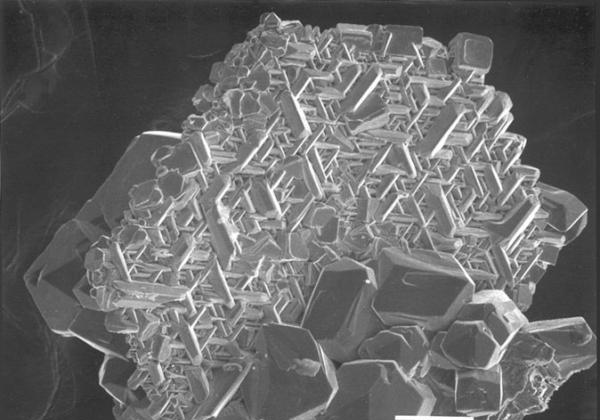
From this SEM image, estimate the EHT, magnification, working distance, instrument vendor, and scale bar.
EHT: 20 kV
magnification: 0.15 K X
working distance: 39 mm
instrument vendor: JEOL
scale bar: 100000 nm